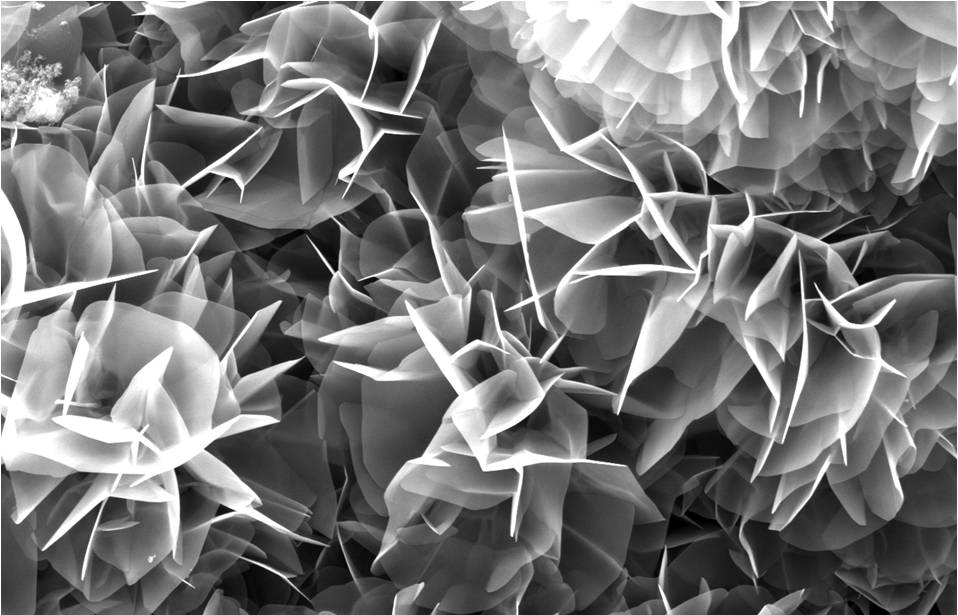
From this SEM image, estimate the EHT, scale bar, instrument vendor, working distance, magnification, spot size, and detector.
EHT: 10 kV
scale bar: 5000 nm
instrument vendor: FEI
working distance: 5.2 mm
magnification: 6.105 K X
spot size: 4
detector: SE